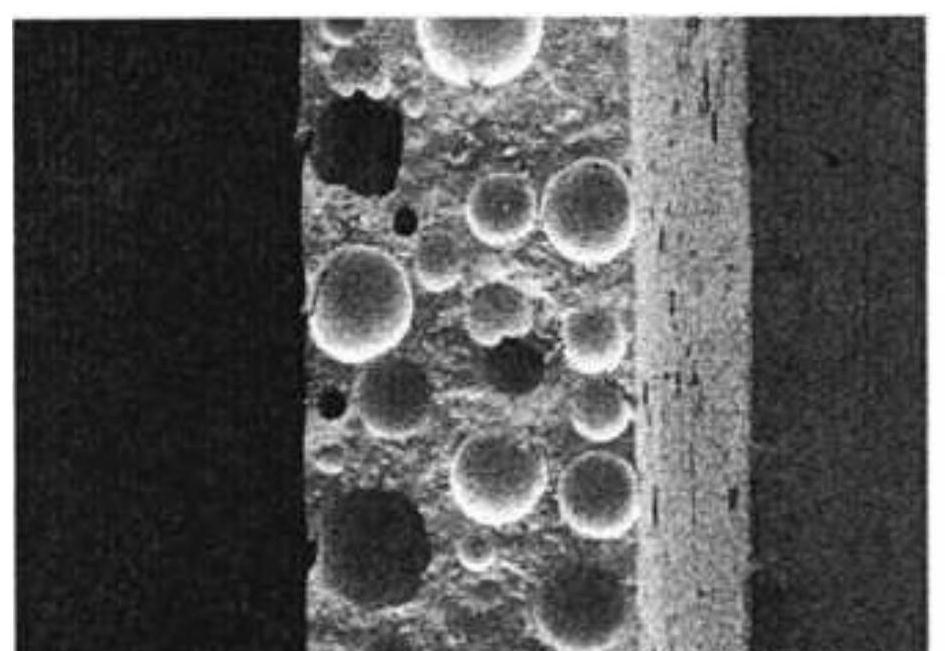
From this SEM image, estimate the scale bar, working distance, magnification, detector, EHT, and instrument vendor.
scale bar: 500000 nm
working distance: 9.1 mm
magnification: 0.1 K X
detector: SE(M)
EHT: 3 kV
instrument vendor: Hitachi